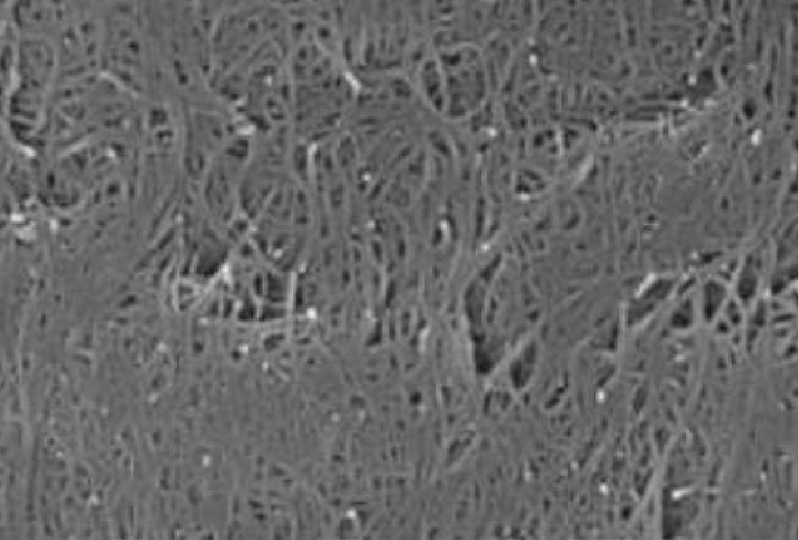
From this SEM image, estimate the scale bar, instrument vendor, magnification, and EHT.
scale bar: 1000 nm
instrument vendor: JEOL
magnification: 25 K X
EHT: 20 kV